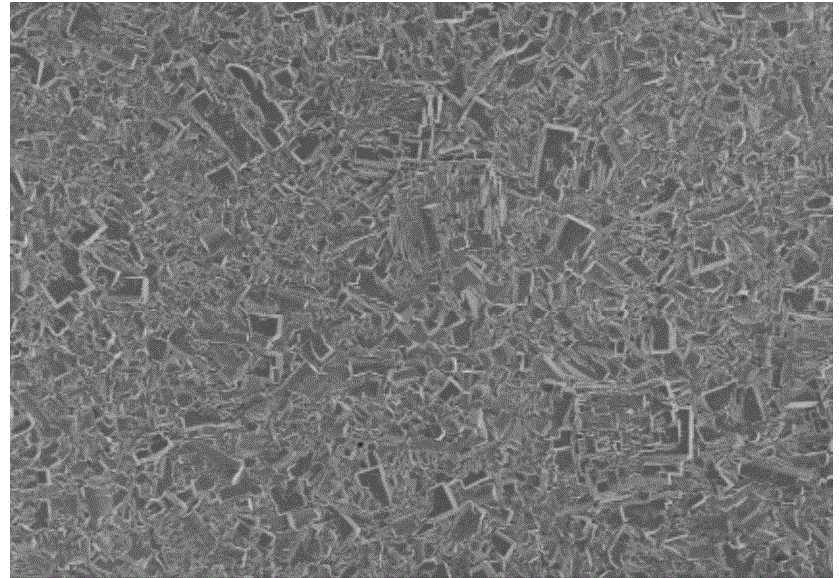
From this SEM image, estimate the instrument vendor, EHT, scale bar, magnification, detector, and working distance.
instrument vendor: Hitachi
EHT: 3 kV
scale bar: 100000 nm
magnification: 0.5 K X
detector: SE(M)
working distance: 8.7 mm